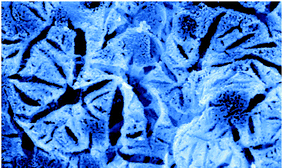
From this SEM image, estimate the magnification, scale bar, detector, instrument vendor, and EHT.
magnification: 4 K X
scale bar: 5000 nm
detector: SEI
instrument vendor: JEOL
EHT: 30 kV